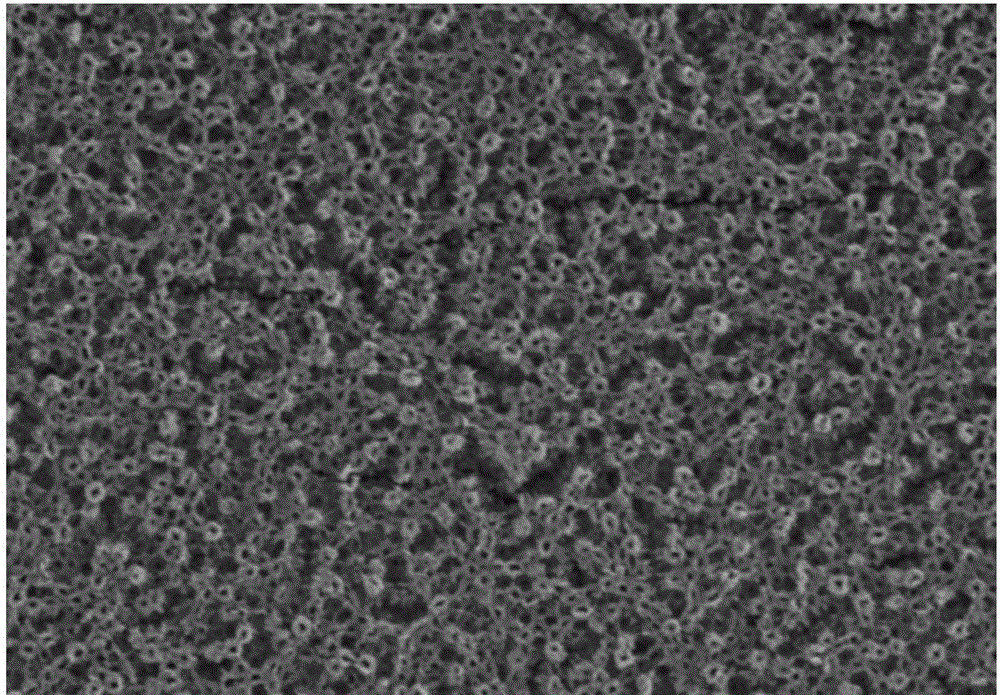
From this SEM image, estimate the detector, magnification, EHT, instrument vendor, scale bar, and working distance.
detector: SE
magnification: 10 K X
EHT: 15 kV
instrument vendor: Hitachi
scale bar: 5000 nm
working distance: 7.2 mm